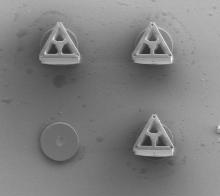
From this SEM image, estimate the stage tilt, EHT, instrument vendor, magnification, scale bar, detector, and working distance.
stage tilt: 0°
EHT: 5 kV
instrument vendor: FEI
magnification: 0.5 K X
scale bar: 200000 nm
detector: ETD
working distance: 8.6 mm